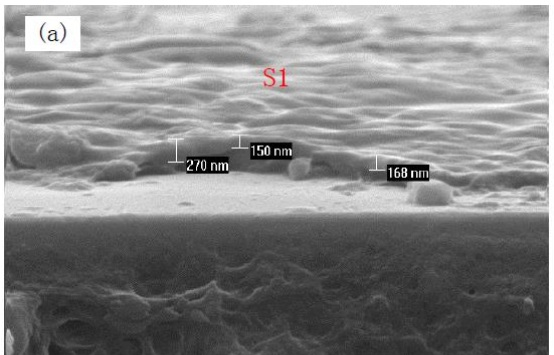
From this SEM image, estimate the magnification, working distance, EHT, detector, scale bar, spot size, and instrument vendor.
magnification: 20 K X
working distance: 10.1 mm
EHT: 10 kV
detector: SE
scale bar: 1000 nm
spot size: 3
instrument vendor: FEI